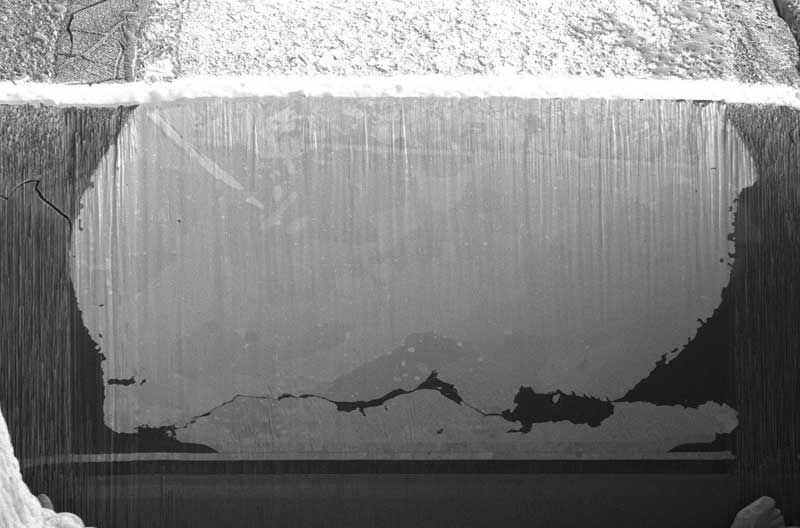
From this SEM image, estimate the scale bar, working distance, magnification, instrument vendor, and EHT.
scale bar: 100000 nm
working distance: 4.2 mm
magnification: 1.2 K X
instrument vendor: FEI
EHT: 2 kV